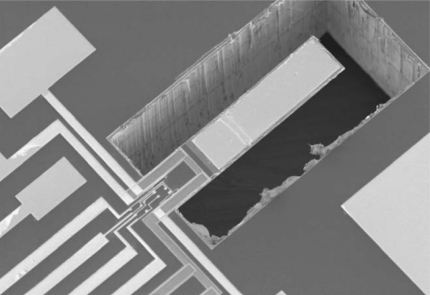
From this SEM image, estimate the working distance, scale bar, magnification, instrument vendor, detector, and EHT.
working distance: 19.1 mm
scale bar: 500000 nm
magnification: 0.09 K X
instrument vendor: JEOL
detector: SE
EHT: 5 kV